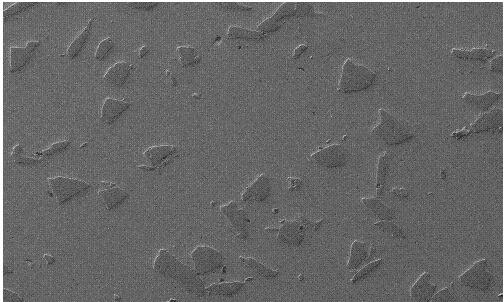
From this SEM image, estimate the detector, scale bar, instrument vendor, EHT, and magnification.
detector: SE1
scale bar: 200000 nm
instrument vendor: Zeiss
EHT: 20 kV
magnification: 0.05 K X